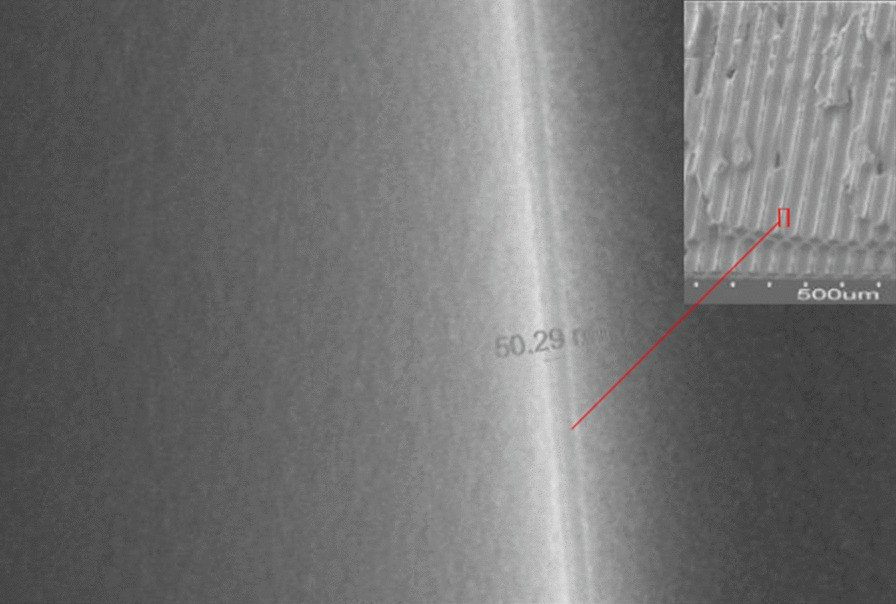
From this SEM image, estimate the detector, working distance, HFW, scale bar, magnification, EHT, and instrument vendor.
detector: ETD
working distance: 14.5 mm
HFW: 2.12 µm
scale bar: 400 nm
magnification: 60 K X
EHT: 10 kV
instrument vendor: FEI